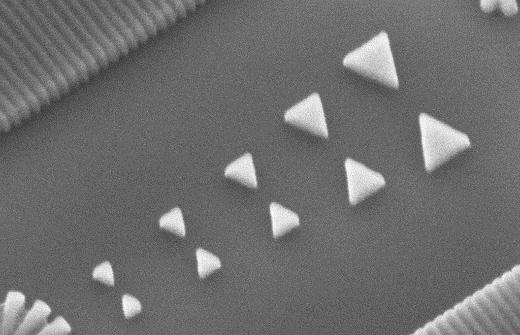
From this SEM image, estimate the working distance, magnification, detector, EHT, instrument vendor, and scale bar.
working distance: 14.7 mm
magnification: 160 K X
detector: SE2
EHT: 5 kV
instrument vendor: Zeiss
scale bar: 200 nm